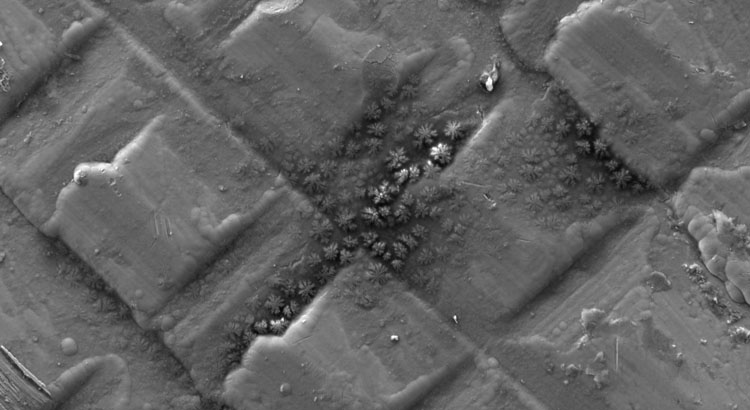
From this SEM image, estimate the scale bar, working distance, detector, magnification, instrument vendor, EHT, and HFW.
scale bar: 50000 nm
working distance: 5.428 mm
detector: SED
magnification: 2.8 K X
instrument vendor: Thermo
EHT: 15 kV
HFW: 248 µm